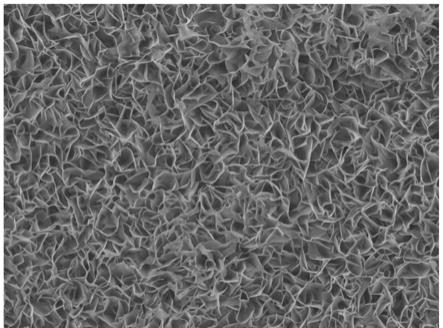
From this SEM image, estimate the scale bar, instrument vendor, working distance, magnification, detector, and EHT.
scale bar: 1000 nm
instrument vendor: JEOL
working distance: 9 mm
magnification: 3.5 K X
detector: LED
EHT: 15 kV